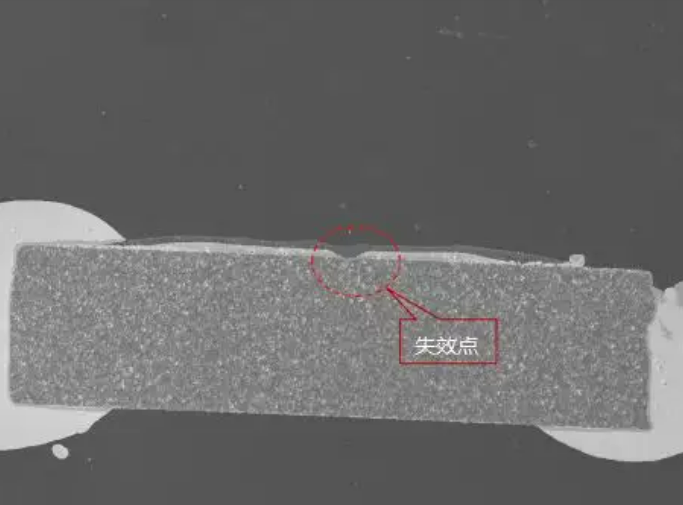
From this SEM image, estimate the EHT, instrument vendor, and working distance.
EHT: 20 kV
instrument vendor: Tescan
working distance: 15.04 mm